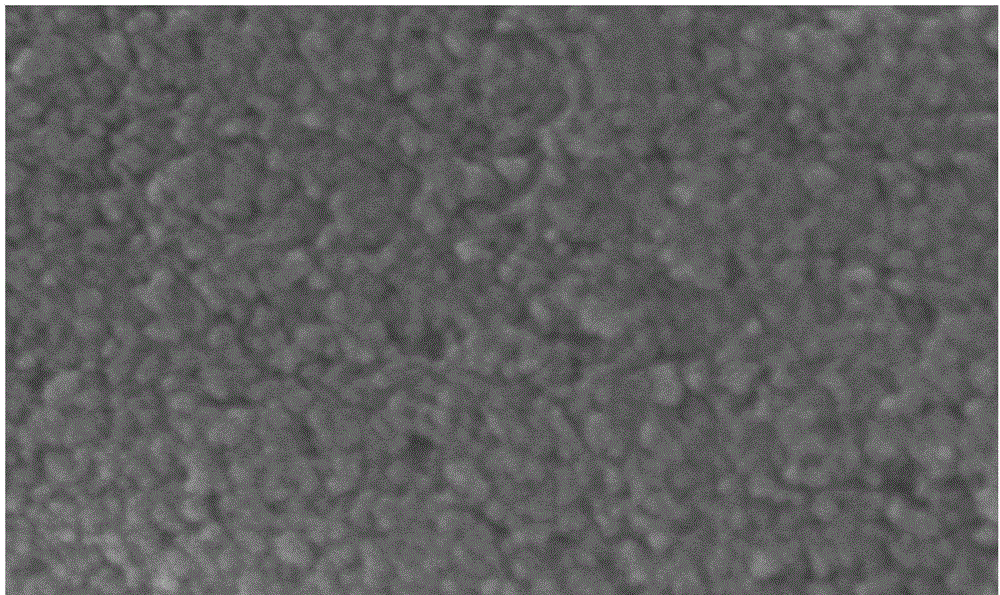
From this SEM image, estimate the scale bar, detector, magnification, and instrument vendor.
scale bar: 500 nm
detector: SE(U)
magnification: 100 K X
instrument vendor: Hitachi